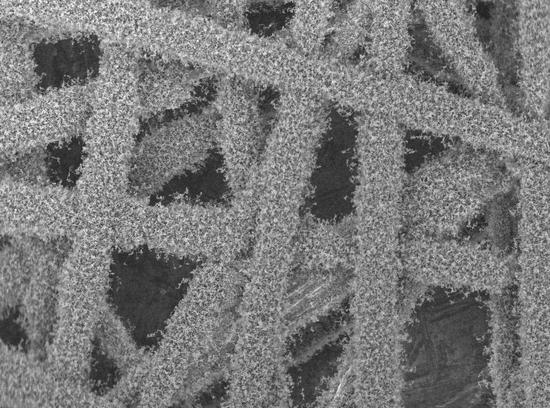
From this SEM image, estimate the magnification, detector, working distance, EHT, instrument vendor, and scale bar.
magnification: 0.25 K X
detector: SEI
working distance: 13.1 mm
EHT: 5 kV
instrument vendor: JEOL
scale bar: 100000 nm